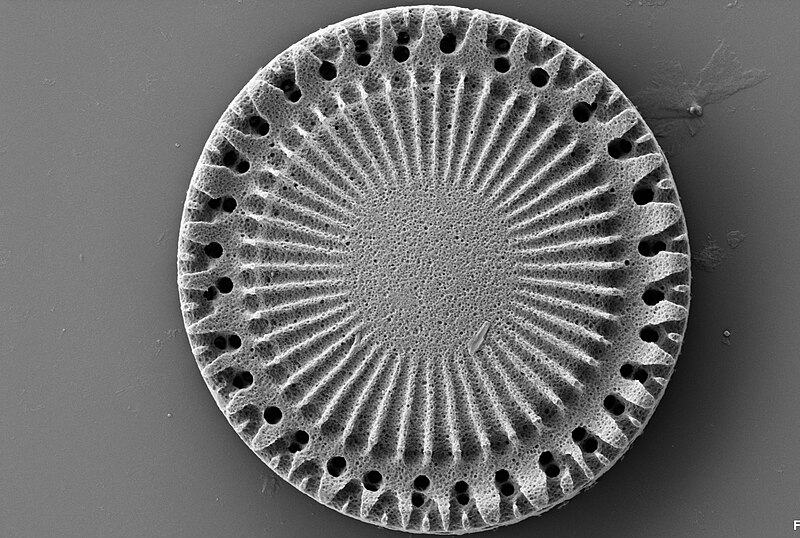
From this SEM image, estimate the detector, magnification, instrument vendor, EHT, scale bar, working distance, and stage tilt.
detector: SE2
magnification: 9.72 K X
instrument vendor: Zeiss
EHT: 3 kV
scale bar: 1000 nm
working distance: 5 mm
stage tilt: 0°